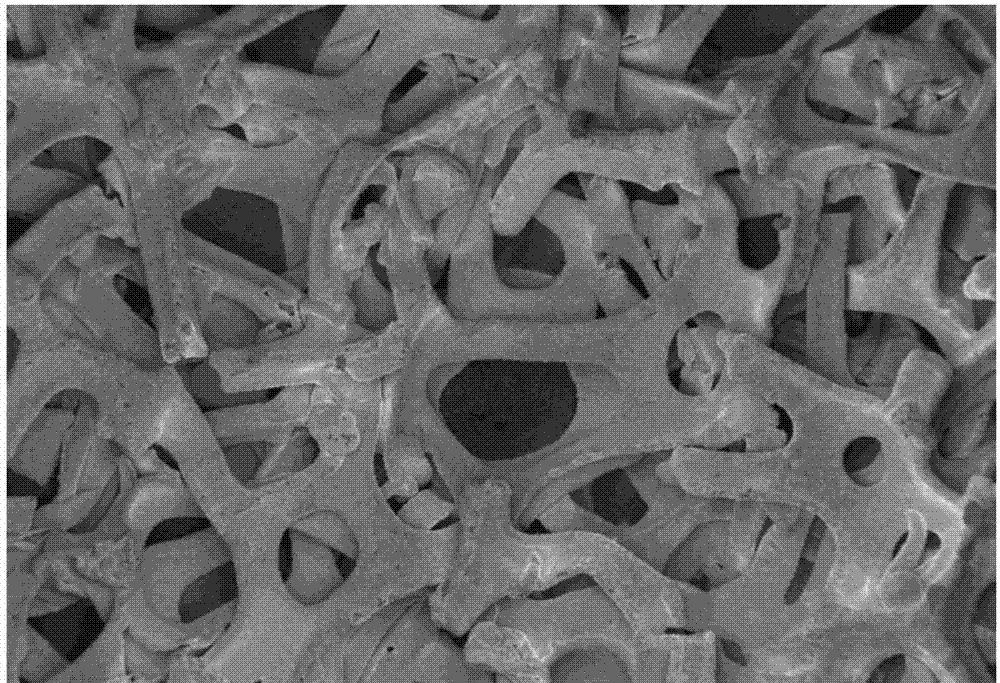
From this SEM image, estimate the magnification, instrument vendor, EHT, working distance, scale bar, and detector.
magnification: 0.2 K X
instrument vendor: Zeiss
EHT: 2 kV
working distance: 11.6 mm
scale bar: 100000 nm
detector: InLens